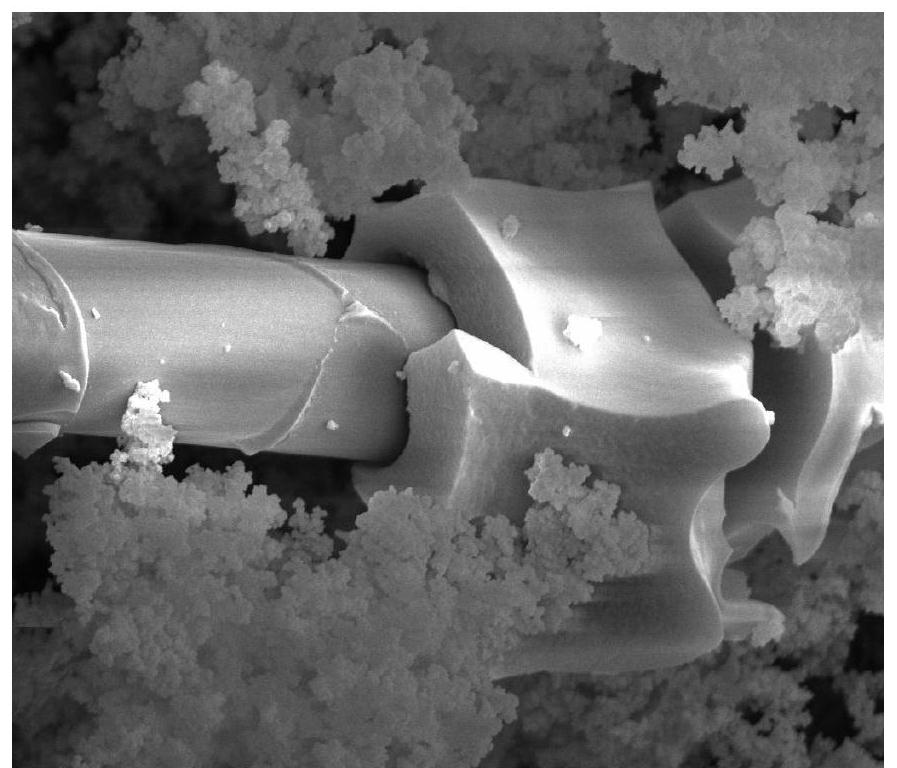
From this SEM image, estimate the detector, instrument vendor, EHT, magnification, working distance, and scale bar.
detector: SE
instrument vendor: FEI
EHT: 20 kV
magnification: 10 K X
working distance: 5.3 mm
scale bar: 5000 nm